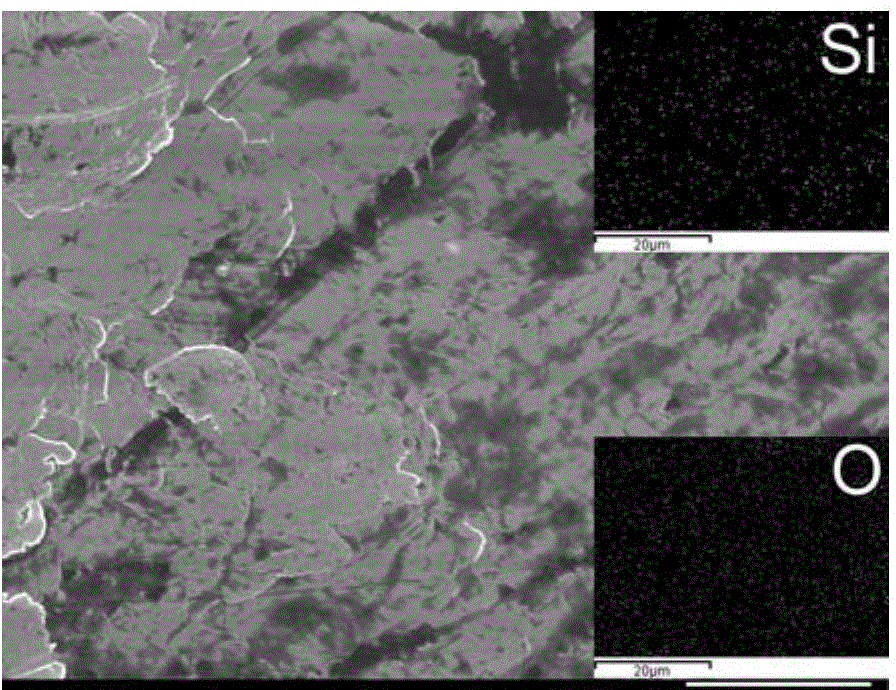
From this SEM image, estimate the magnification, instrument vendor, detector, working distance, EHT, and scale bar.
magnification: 2.5 K X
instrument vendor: JEOL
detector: SEI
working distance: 14.7 mm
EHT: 15 kV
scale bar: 10000 nm